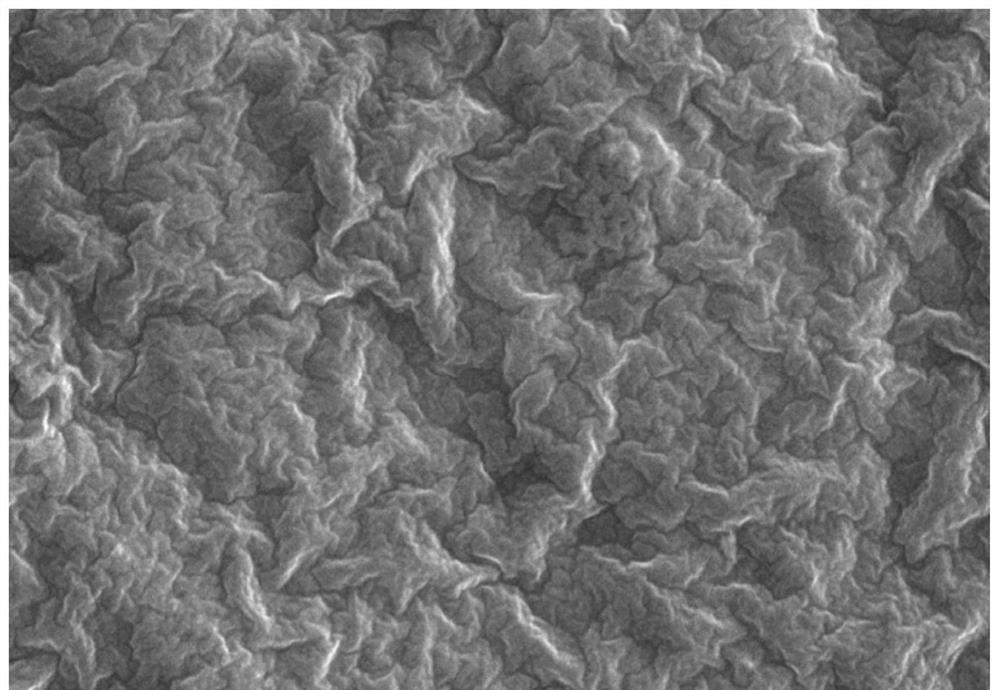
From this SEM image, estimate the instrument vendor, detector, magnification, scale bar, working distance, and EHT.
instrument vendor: Hitachi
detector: SE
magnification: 5 K X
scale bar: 10000 nm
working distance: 5.5 mm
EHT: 10 kV